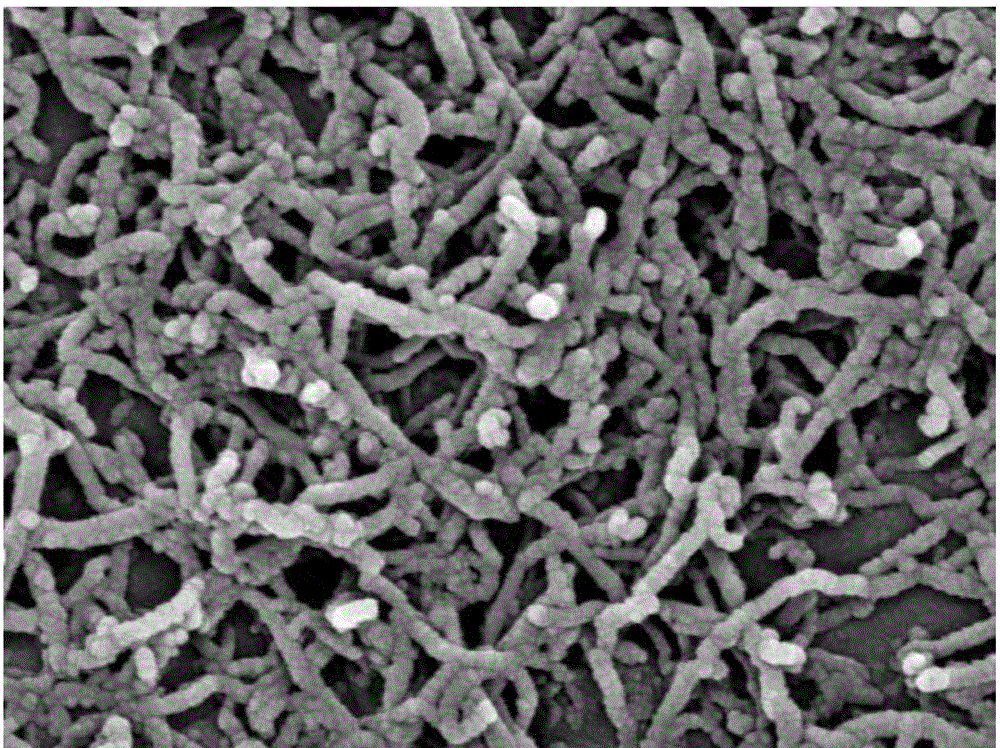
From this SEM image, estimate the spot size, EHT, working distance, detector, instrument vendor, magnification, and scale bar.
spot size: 5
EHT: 20 kV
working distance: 5 mm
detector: TLD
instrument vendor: FEI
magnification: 40 K X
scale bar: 500 nm